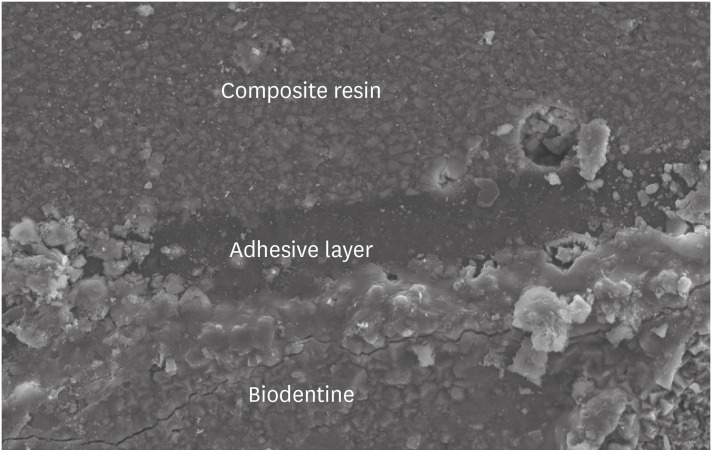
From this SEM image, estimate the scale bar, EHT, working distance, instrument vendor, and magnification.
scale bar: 10000 nm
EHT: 15 kV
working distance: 9 mm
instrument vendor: JEOL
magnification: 1 K X